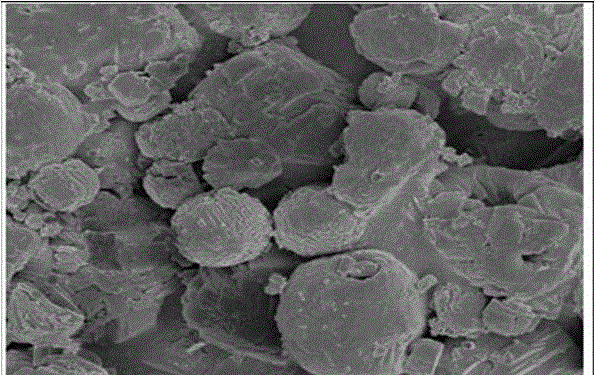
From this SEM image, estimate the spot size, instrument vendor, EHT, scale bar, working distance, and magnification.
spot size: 3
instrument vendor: FEI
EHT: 3 kV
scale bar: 3000 nm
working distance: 5.3 mm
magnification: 30 K X